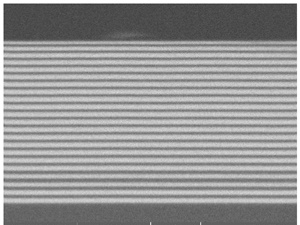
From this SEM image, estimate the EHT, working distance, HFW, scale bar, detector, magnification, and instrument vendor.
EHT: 9 kV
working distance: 16 mm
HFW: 5.84 µm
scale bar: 1000 nm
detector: BSE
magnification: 32.4 K X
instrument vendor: Tescan